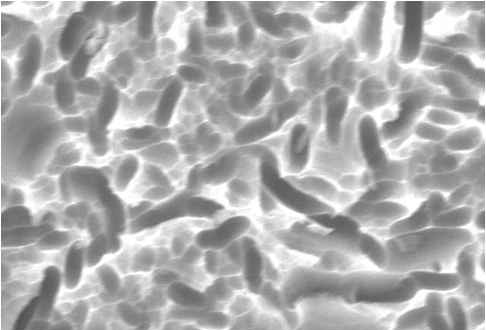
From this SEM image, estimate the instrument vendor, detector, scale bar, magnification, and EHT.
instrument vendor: JEOL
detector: SEI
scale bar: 5000 nm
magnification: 3 K X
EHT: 20 kV